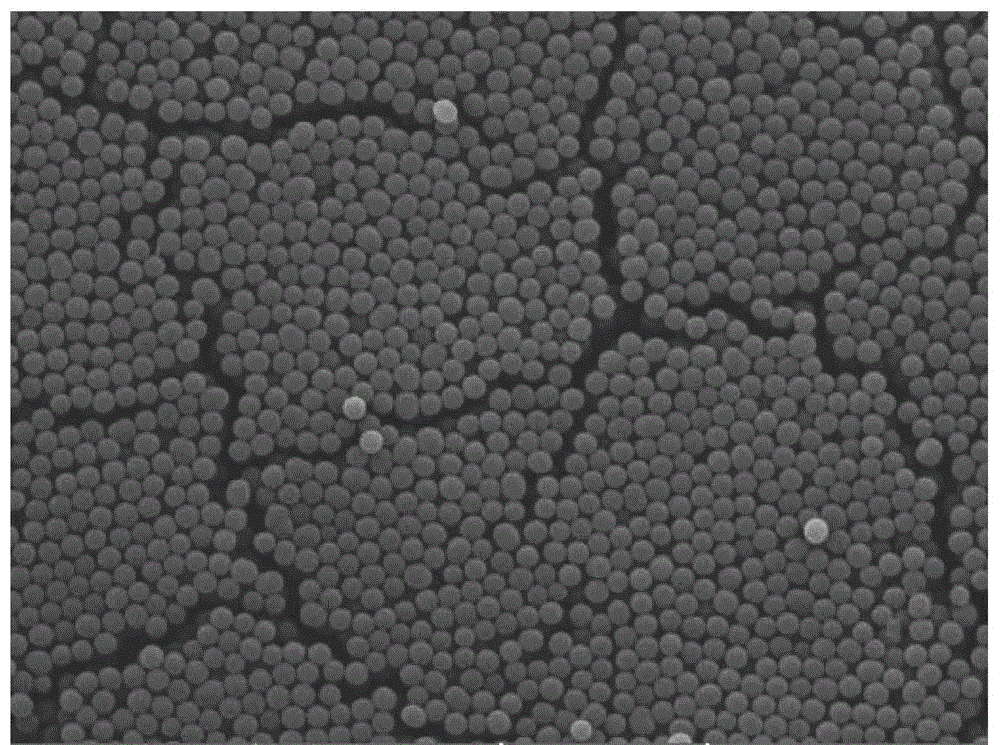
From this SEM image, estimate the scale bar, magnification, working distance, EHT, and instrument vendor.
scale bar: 5000 nm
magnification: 6.27 K X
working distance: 5.09 mm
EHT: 15 kV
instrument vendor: Tescan